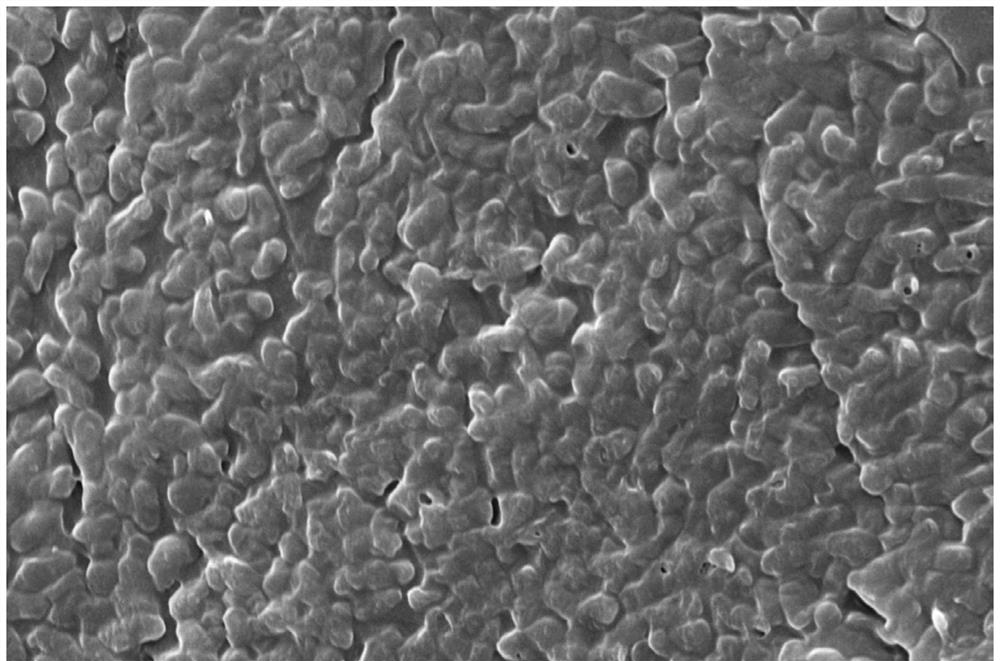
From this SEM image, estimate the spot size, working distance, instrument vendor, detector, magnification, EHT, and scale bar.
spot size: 3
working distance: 8.1 mm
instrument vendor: FEI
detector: ETD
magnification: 20 K X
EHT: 10 kV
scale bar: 5000 nm